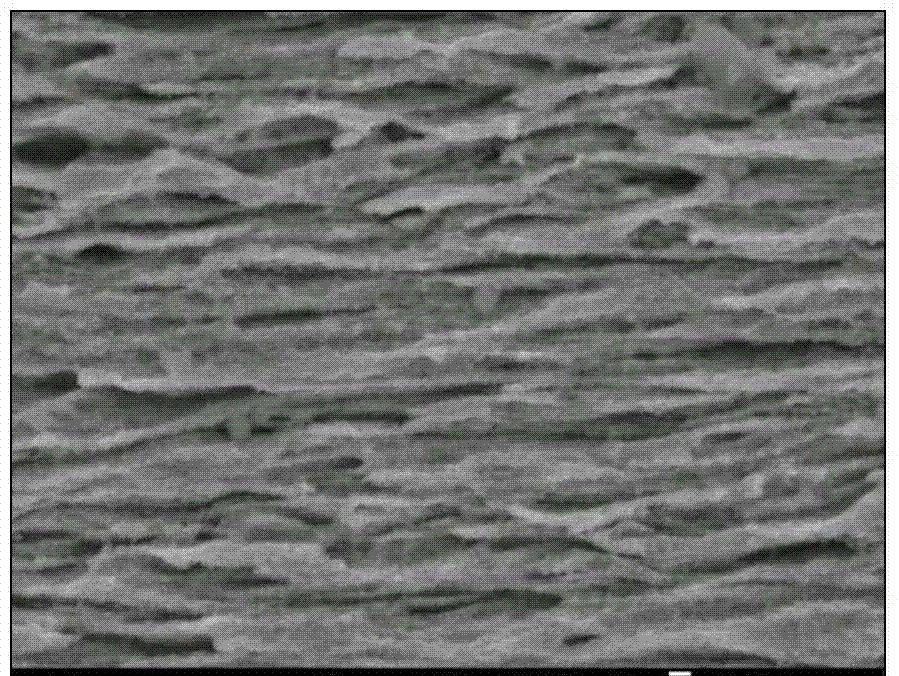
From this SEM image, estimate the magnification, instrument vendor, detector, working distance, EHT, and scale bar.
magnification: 30 K X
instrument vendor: JEOL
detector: SEI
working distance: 8.3 mm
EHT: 5 kV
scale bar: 100 nm